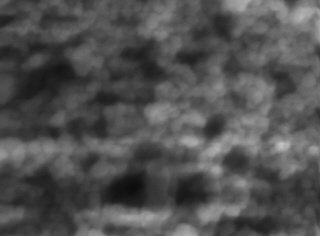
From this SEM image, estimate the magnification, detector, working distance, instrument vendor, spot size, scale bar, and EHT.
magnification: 40 K X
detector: TLD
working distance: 5.4 mm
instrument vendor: FEI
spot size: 5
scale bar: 500 nm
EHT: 10 kV